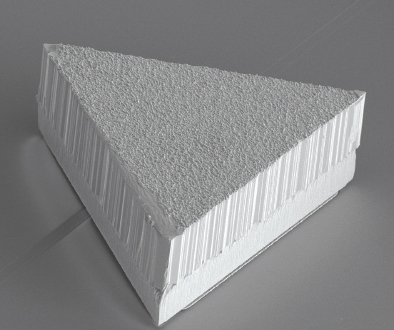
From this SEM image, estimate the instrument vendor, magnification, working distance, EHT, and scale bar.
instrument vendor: FEI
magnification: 0.6 K X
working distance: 7.5 mm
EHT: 10 kV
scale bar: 300000 nm